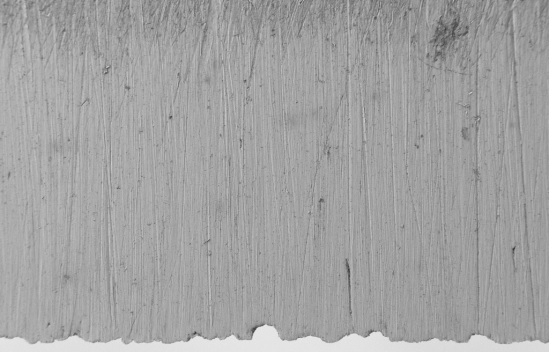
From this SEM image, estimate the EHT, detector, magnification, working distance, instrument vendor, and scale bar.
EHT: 1 kV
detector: SE2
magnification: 0.15 K X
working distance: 4.4 mm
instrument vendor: Zeiss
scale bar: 100000 nm